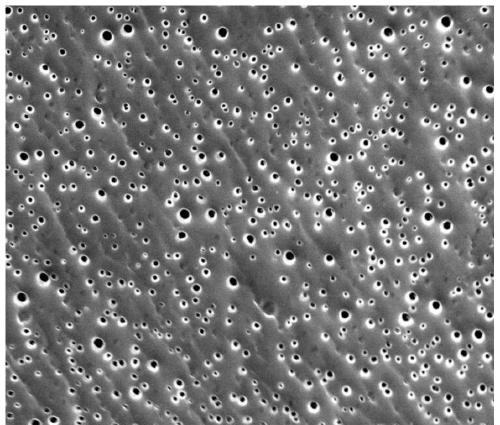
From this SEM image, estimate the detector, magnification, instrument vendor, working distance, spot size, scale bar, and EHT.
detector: TLD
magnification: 20 K X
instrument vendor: FEI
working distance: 4.7 mm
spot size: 3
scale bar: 5000 nm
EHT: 10 kV